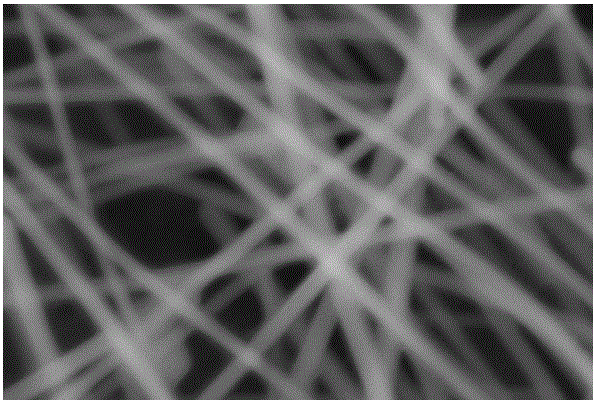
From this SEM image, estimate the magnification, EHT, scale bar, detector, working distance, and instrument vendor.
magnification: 100 K X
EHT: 10 kV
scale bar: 100 nm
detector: SE2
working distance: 4.7 mm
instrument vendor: Zeiss